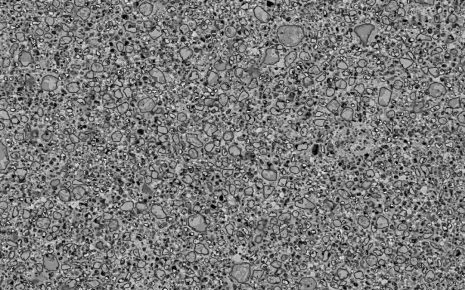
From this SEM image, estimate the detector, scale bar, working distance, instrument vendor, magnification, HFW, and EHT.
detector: BSED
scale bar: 50000 nm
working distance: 9.7 mm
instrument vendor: FEI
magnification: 2 K X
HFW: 207 µm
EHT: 20 kV